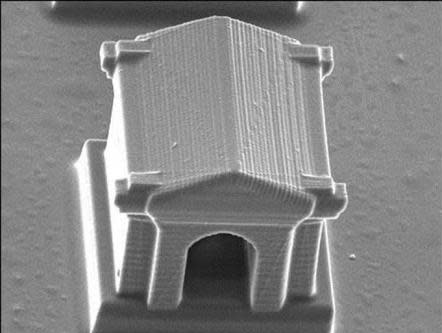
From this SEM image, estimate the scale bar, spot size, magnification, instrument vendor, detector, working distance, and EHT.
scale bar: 20000 nm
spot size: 4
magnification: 0.746 K X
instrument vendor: FEI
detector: SE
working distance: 27.4 mm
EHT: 10 kV